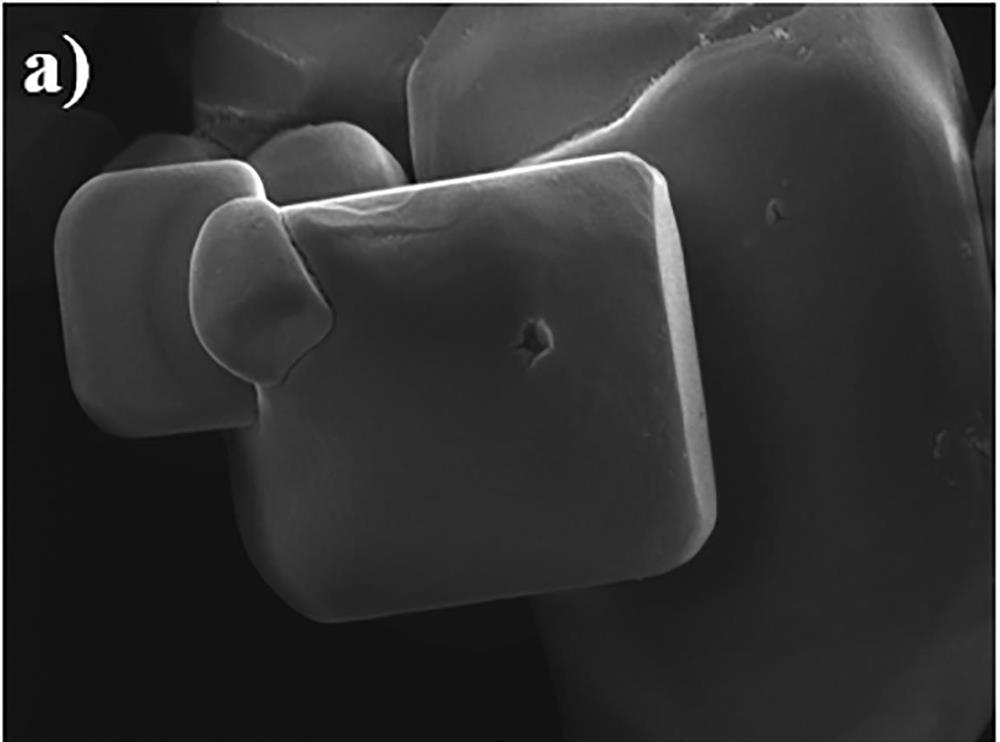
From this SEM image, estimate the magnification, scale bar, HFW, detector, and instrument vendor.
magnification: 5 K X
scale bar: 10000 nm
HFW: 55.4 µm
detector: SE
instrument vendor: Tescan